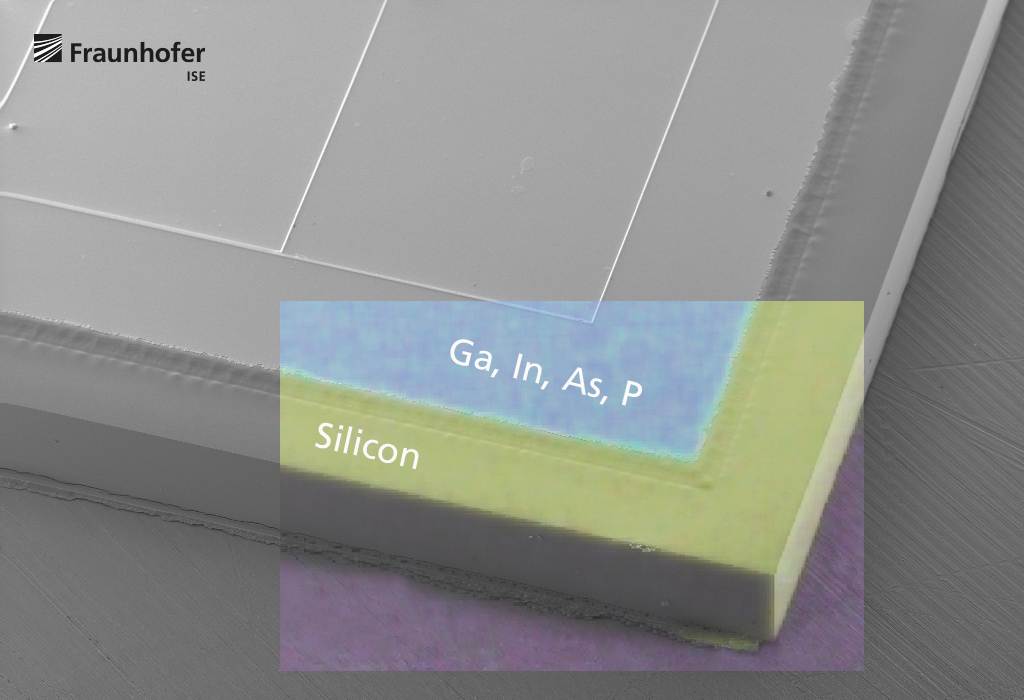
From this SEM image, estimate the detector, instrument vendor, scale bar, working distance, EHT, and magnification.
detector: SE2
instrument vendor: Zeiss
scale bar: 100000 nm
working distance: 5.7 mm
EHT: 10 kV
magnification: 0.042 K X